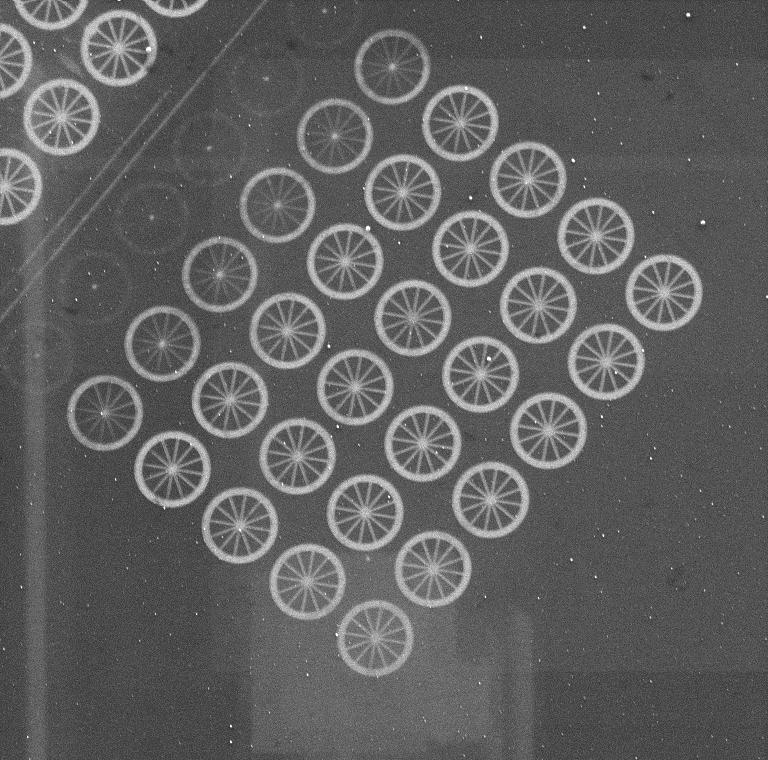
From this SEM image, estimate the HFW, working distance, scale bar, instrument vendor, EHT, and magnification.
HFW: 60.12 µm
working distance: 3.794 mm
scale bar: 10000 nm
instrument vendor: Tescan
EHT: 2 kV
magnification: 3.6 K X